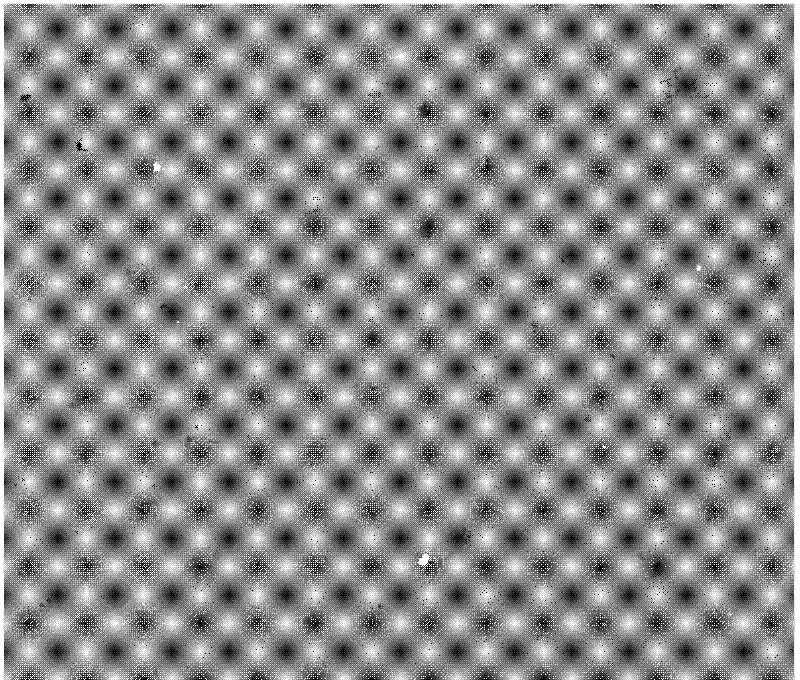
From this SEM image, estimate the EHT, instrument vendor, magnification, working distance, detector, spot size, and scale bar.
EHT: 20 kV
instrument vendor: FEI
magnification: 2 K X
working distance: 9.7 mm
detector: ETD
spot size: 4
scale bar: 20000 nm